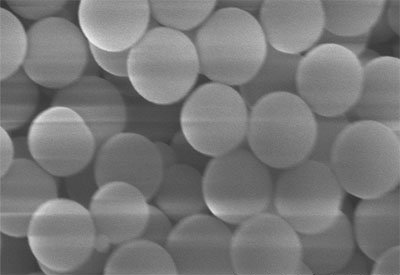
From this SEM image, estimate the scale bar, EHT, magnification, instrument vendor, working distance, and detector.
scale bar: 1000 nm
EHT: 5 kV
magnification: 50 K X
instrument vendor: Hitachi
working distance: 8.9 mm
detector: SE(UL)